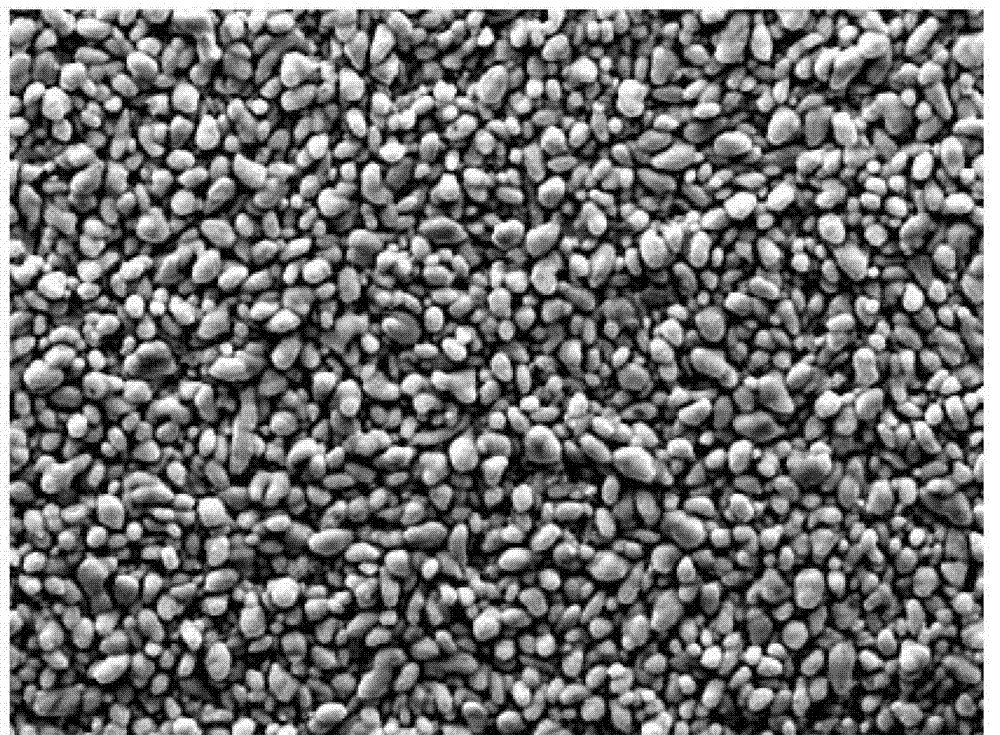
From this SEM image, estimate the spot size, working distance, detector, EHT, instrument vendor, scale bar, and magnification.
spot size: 5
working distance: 15.3 mm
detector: SE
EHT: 20 kV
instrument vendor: FEI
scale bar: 10000 nm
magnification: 2 K X